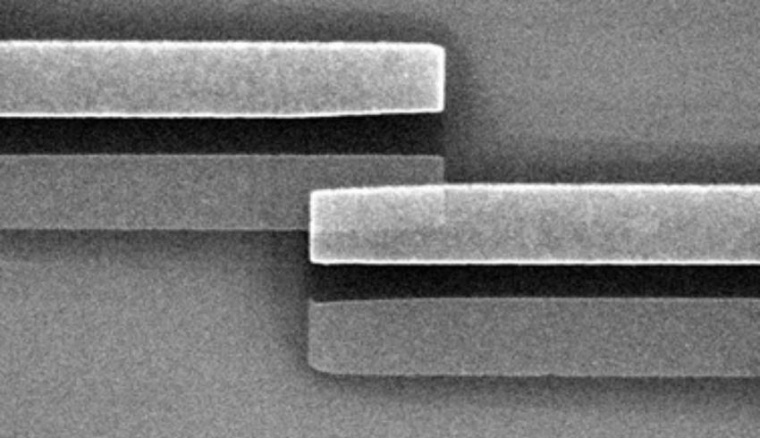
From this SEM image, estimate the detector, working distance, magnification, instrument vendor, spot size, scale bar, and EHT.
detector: SE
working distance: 17 mm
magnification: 16.366 K X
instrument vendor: FEI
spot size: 3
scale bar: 2000 nm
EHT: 10 kV